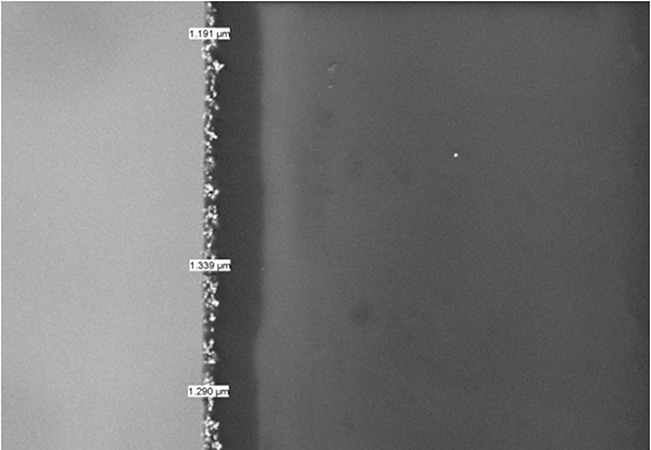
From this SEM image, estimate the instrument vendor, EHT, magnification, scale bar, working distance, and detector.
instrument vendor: Hitachi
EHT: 10 kV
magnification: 2 K X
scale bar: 20000 nm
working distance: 9.6 mm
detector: BSE3D